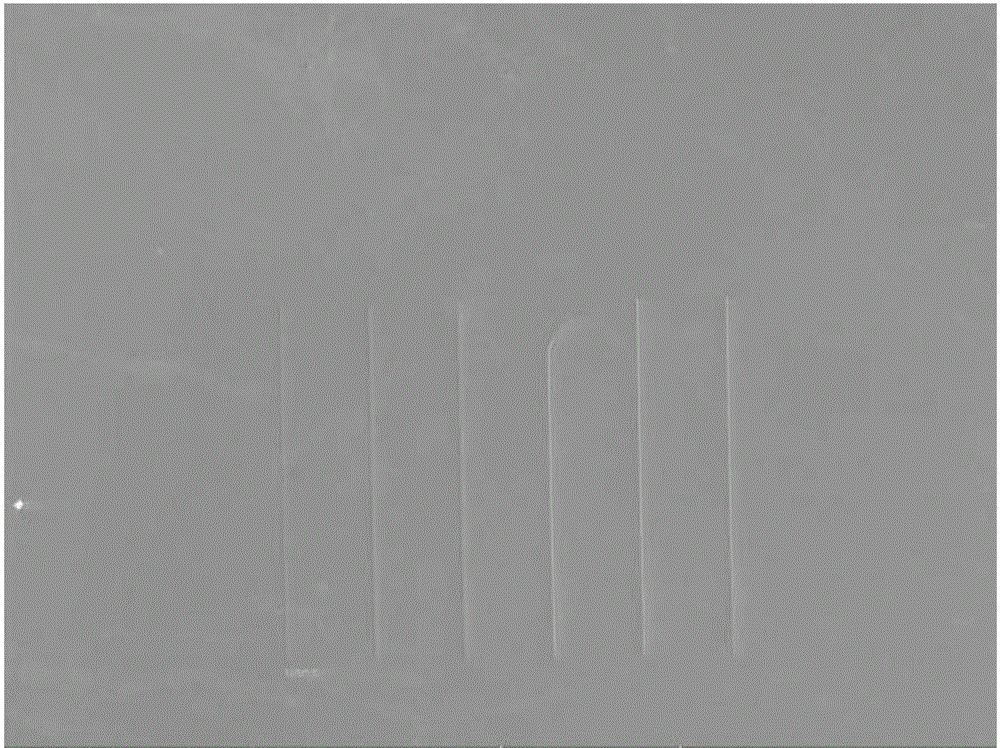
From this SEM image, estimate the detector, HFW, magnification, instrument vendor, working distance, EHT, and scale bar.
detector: SE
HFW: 553 µm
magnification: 0.5 K X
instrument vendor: Tescan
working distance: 8.98 mm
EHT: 15 kV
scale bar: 100000 nm